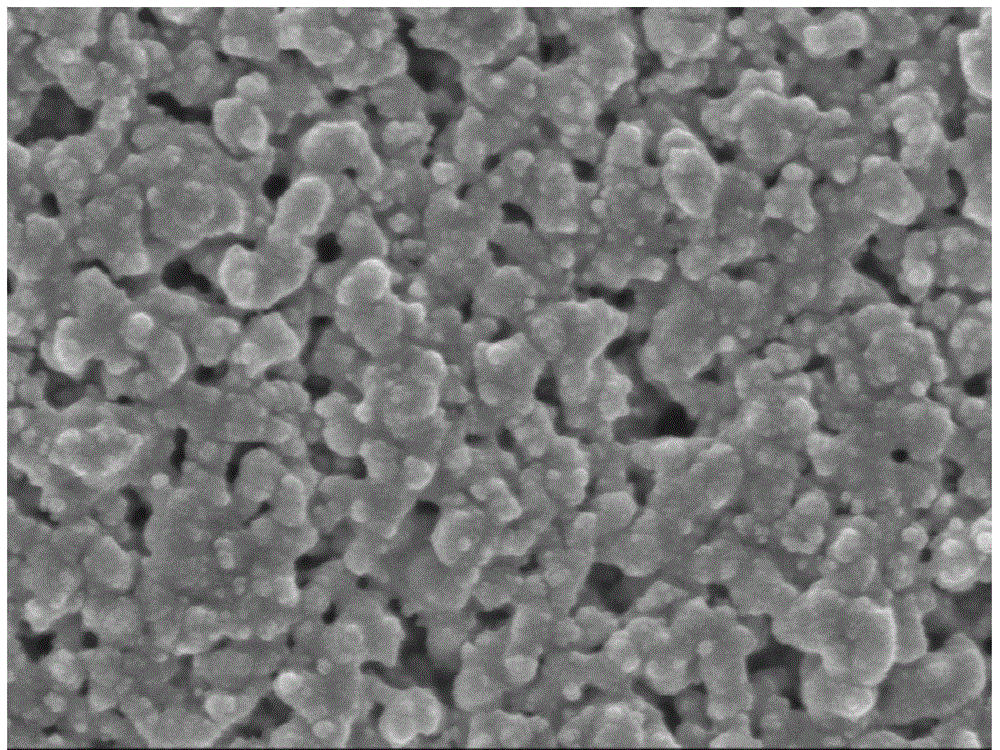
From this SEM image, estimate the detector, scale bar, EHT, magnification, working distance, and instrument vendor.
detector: SEI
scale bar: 100 nm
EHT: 3 kV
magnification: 60 K X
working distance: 5.2 mm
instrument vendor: JEOL